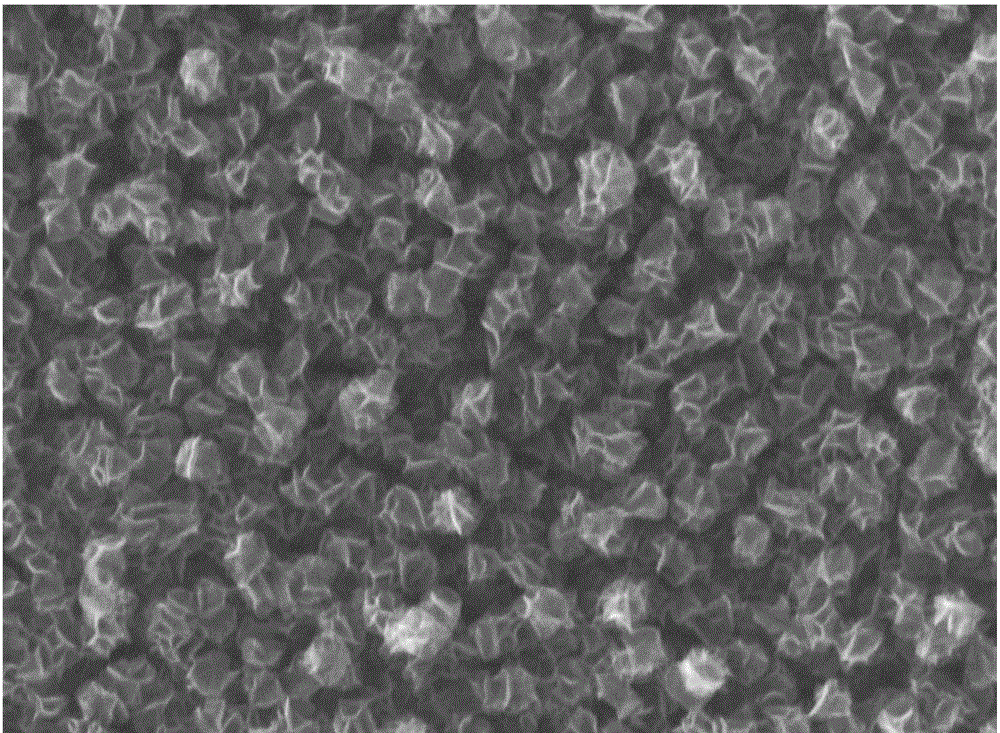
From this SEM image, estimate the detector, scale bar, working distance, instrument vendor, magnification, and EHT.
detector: InLens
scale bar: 200 nm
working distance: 6.3 mm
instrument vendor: Zeiss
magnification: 50 K X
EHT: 10 kV